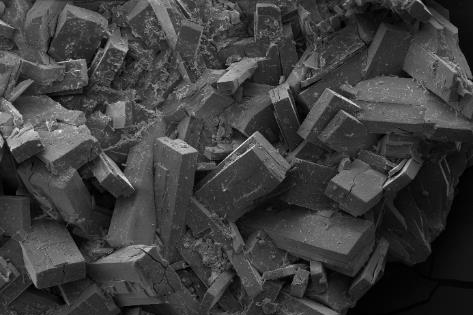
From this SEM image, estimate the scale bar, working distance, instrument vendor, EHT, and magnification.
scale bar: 200000 nm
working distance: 12.5 mm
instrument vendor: Zeiss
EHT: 10 kV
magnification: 0.136 K X